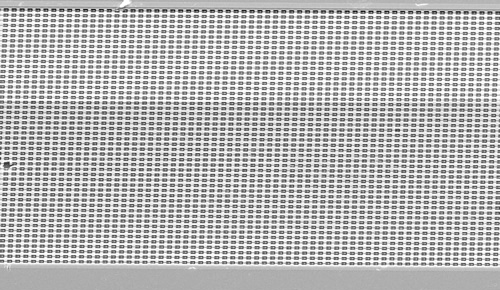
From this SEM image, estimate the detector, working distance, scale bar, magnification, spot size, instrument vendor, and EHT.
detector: SE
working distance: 11.2 mm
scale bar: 50000 nm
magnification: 0.4 K X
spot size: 3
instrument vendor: FEI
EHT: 20 kV